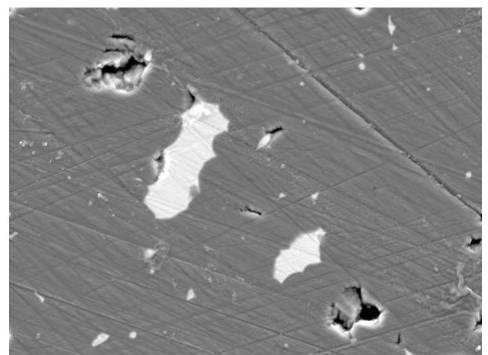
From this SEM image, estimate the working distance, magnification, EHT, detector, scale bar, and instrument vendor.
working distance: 10 mm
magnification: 3 K X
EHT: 10 kV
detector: SEI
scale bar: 1000 nm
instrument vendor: JEOL